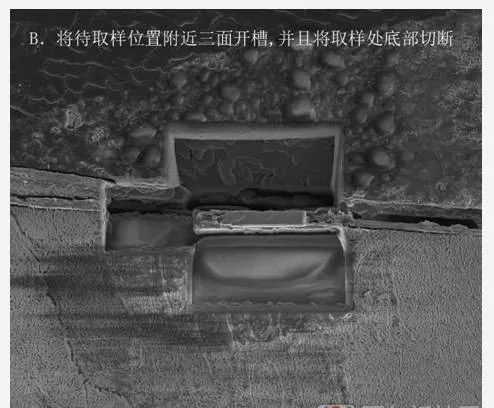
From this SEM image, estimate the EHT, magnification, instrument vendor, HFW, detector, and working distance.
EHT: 5 kV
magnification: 5 K X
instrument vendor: FEI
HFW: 51.2 µm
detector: ETD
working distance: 4 mm